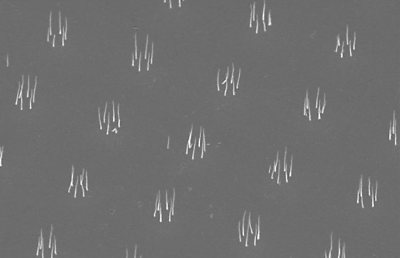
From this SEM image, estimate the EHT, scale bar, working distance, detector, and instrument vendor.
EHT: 15 kV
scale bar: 2000 nm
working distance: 8 mm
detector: SE1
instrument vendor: Zeiss